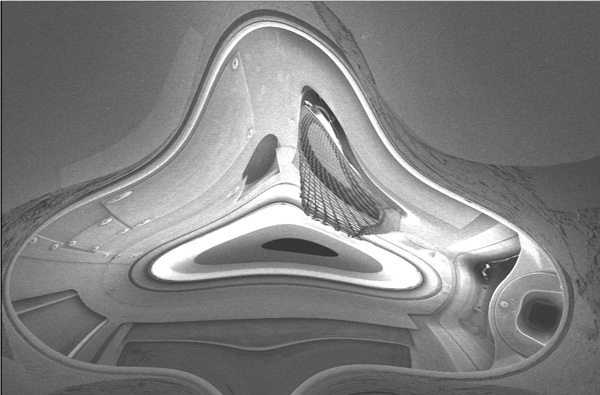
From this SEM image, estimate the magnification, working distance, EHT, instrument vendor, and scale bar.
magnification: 0.025 K X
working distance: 39 mm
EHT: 2 kV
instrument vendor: JEOL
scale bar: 1000000 nm